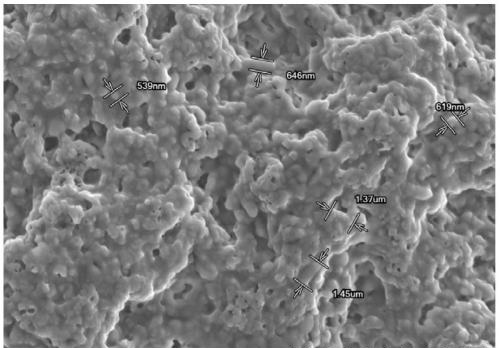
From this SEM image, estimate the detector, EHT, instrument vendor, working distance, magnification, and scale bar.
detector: SE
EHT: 5 kV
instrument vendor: Hitachi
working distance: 6.5 mm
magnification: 5 K X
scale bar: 10000 nm